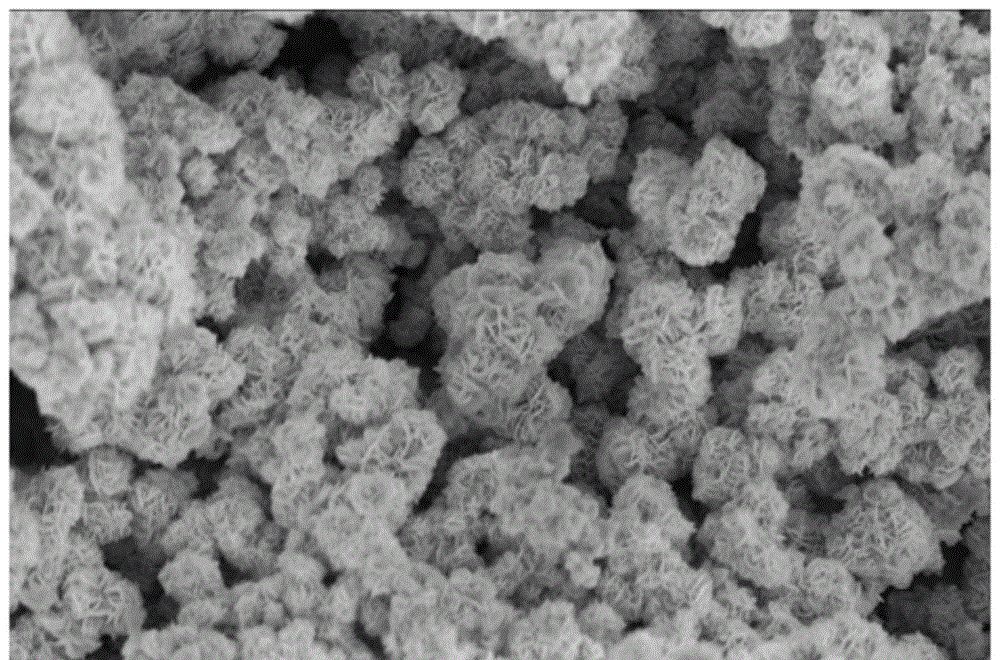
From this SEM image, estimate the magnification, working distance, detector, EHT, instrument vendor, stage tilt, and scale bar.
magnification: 35 K X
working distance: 4.6 mm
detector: TLD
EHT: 3 kV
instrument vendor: FEI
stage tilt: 0°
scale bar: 3000 nm